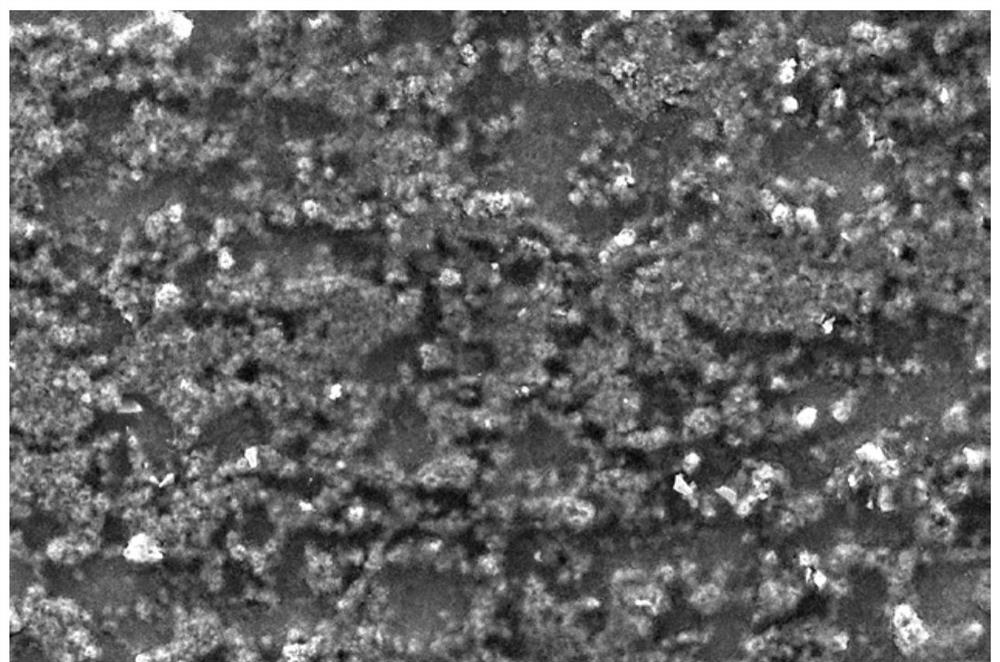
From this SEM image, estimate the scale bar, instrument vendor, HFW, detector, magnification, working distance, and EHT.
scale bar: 100000 nm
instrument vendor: FEI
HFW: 345 µm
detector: ETD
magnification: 1.2 K X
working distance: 10.6 mm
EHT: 20 kV